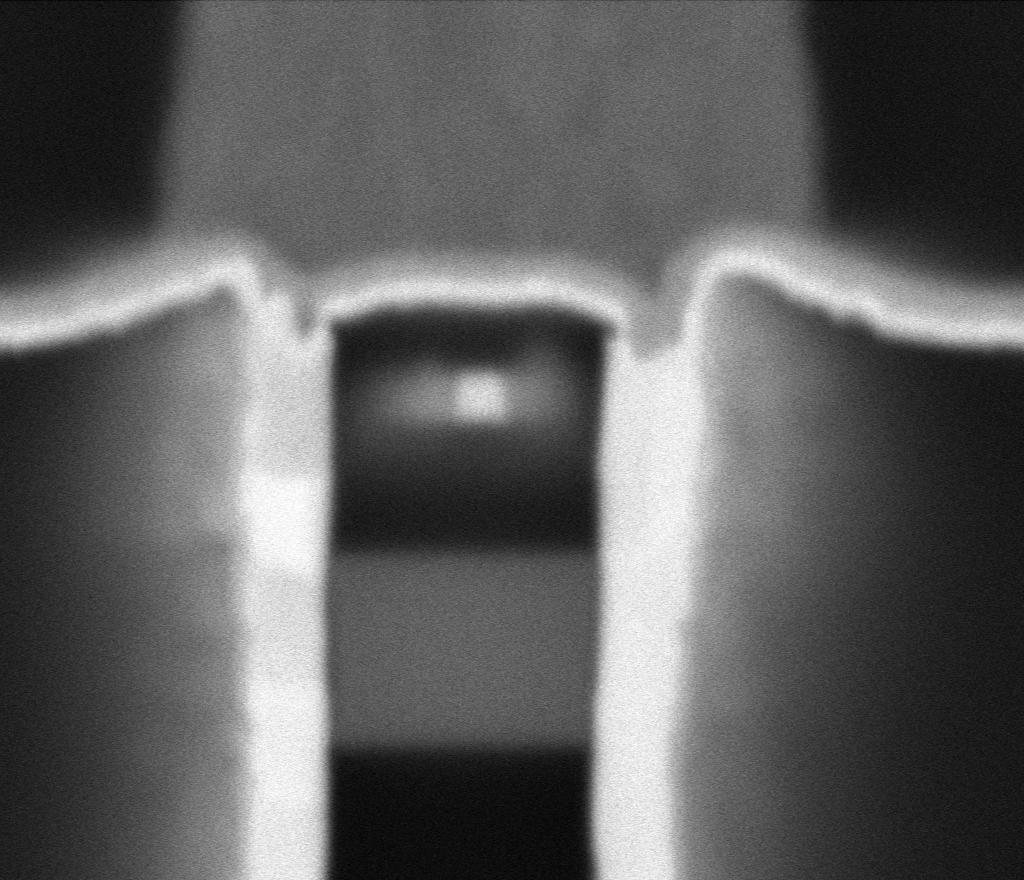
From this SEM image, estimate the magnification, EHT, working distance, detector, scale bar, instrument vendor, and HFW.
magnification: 568.609 K X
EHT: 5 kV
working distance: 2 mm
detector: MD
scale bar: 100 nm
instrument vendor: Thermo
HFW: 0.635 µm